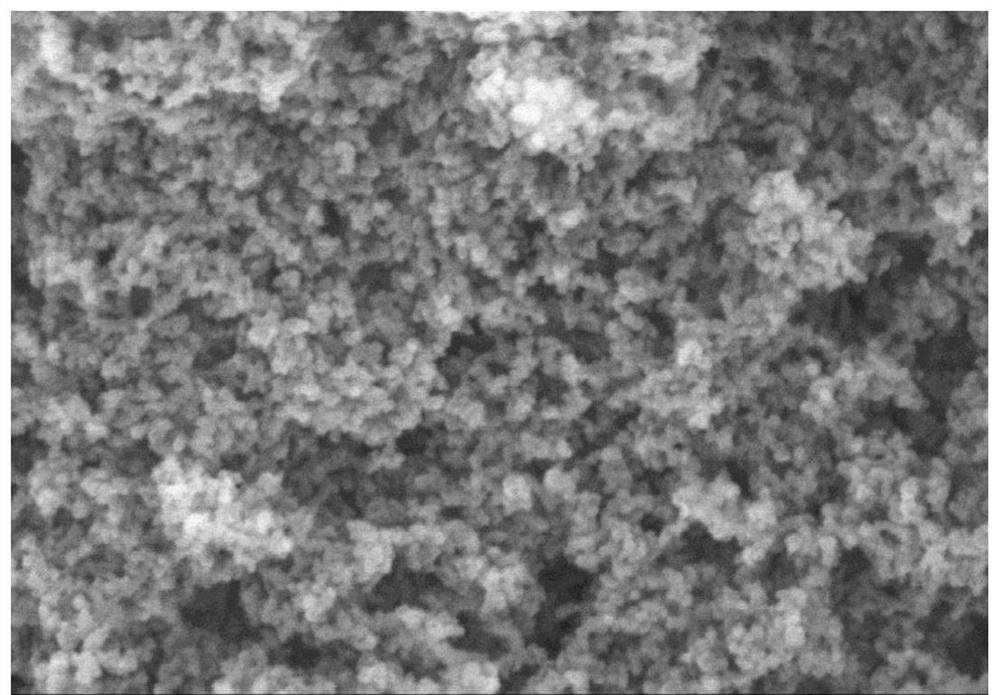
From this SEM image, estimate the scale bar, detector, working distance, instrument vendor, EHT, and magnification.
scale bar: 1000 nm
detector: SE(M)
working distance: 15.2 mm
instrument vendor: Hitachi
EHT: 15 kV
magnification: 50 K X